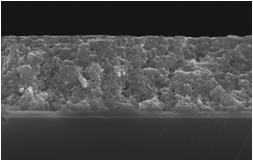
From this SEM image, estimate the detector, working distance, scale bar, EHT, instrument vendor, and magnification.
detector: SE(U)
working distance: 7.6 mm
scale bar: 1000 nm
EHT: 10 kV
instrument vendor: Hitachi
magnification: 30 K X